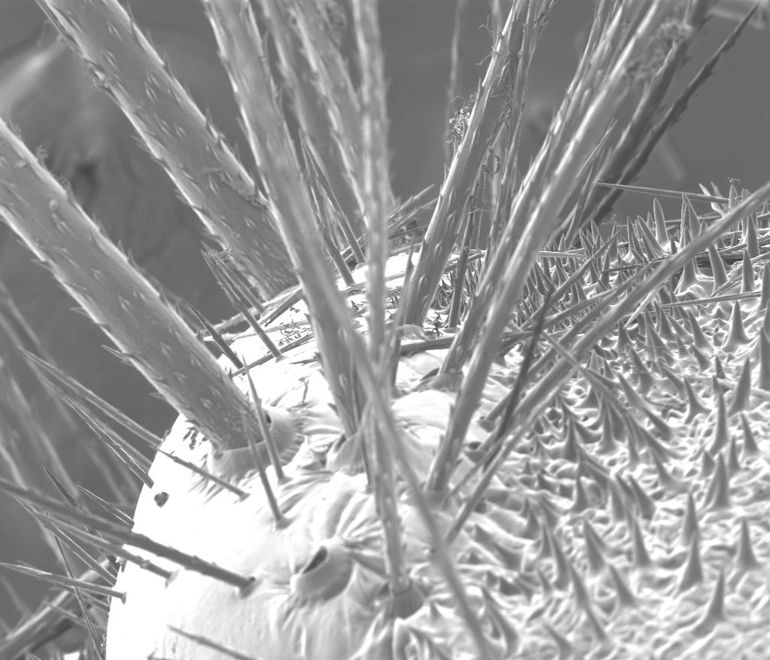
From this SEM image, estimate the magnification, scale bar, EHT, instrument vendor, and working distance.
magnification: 0.243 K X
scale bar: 200000 nm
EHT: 30 kV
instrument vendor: FEI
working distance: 18.5 mm